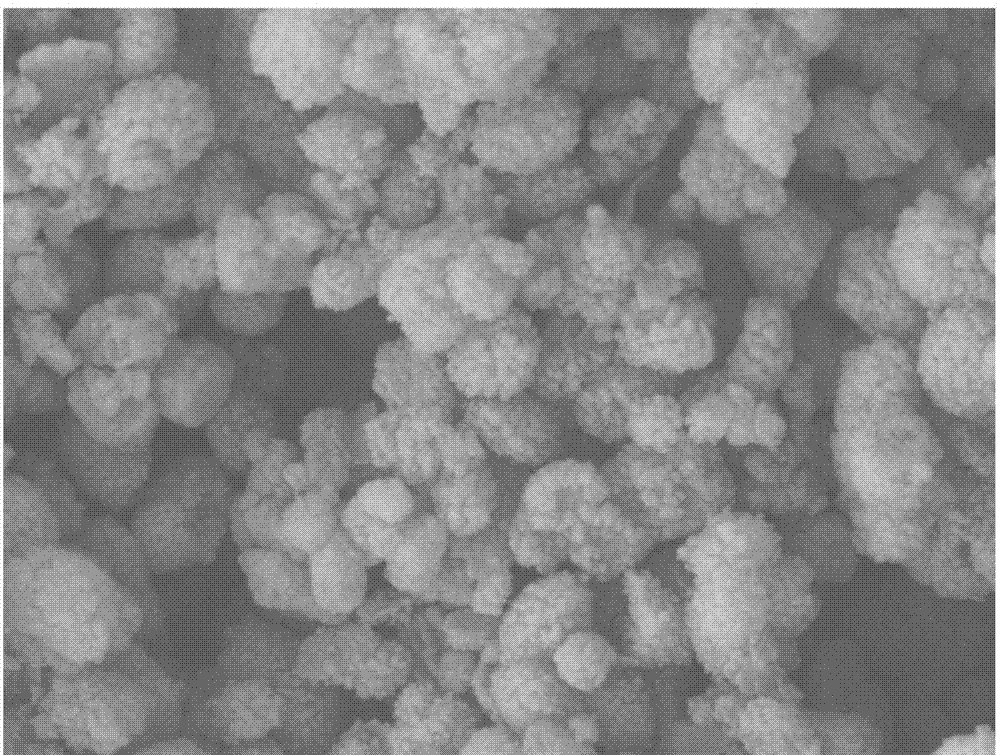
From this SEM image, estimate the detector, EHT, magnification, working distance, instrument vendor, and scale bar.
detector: LEI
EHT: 5 kV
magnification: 30 K X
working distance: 8.1 mm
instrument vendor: JEOL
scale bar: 100 nm